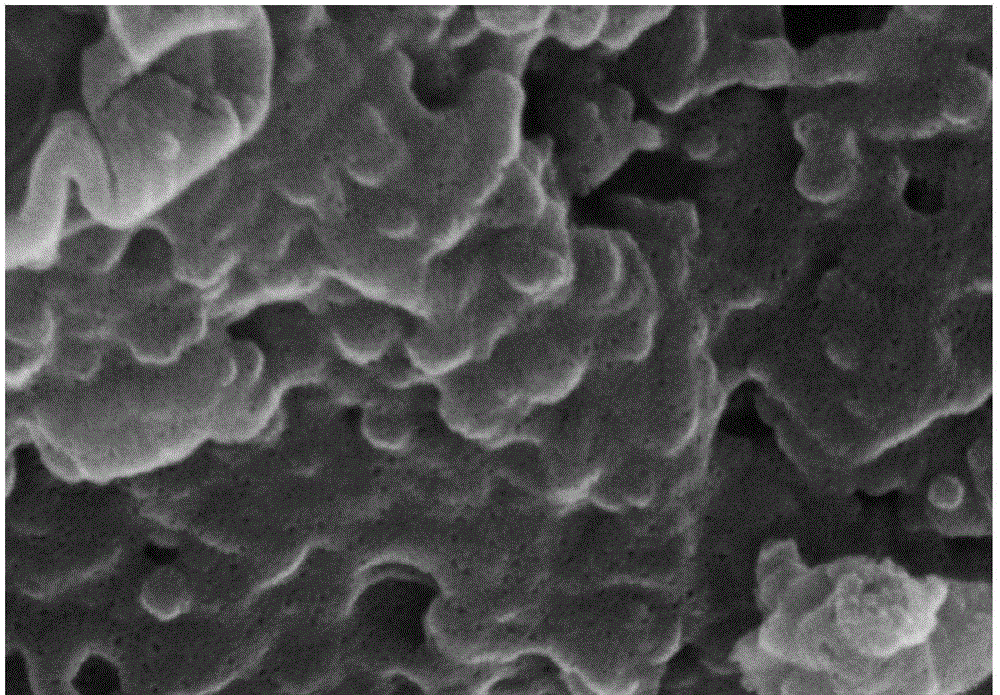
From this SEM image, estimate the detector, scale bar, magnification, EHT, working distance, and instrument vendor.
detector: SE(U,LA0)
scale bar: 500 nm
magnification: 90 K X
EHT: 10 kV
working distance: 8.7 mm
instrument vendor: Hitachi